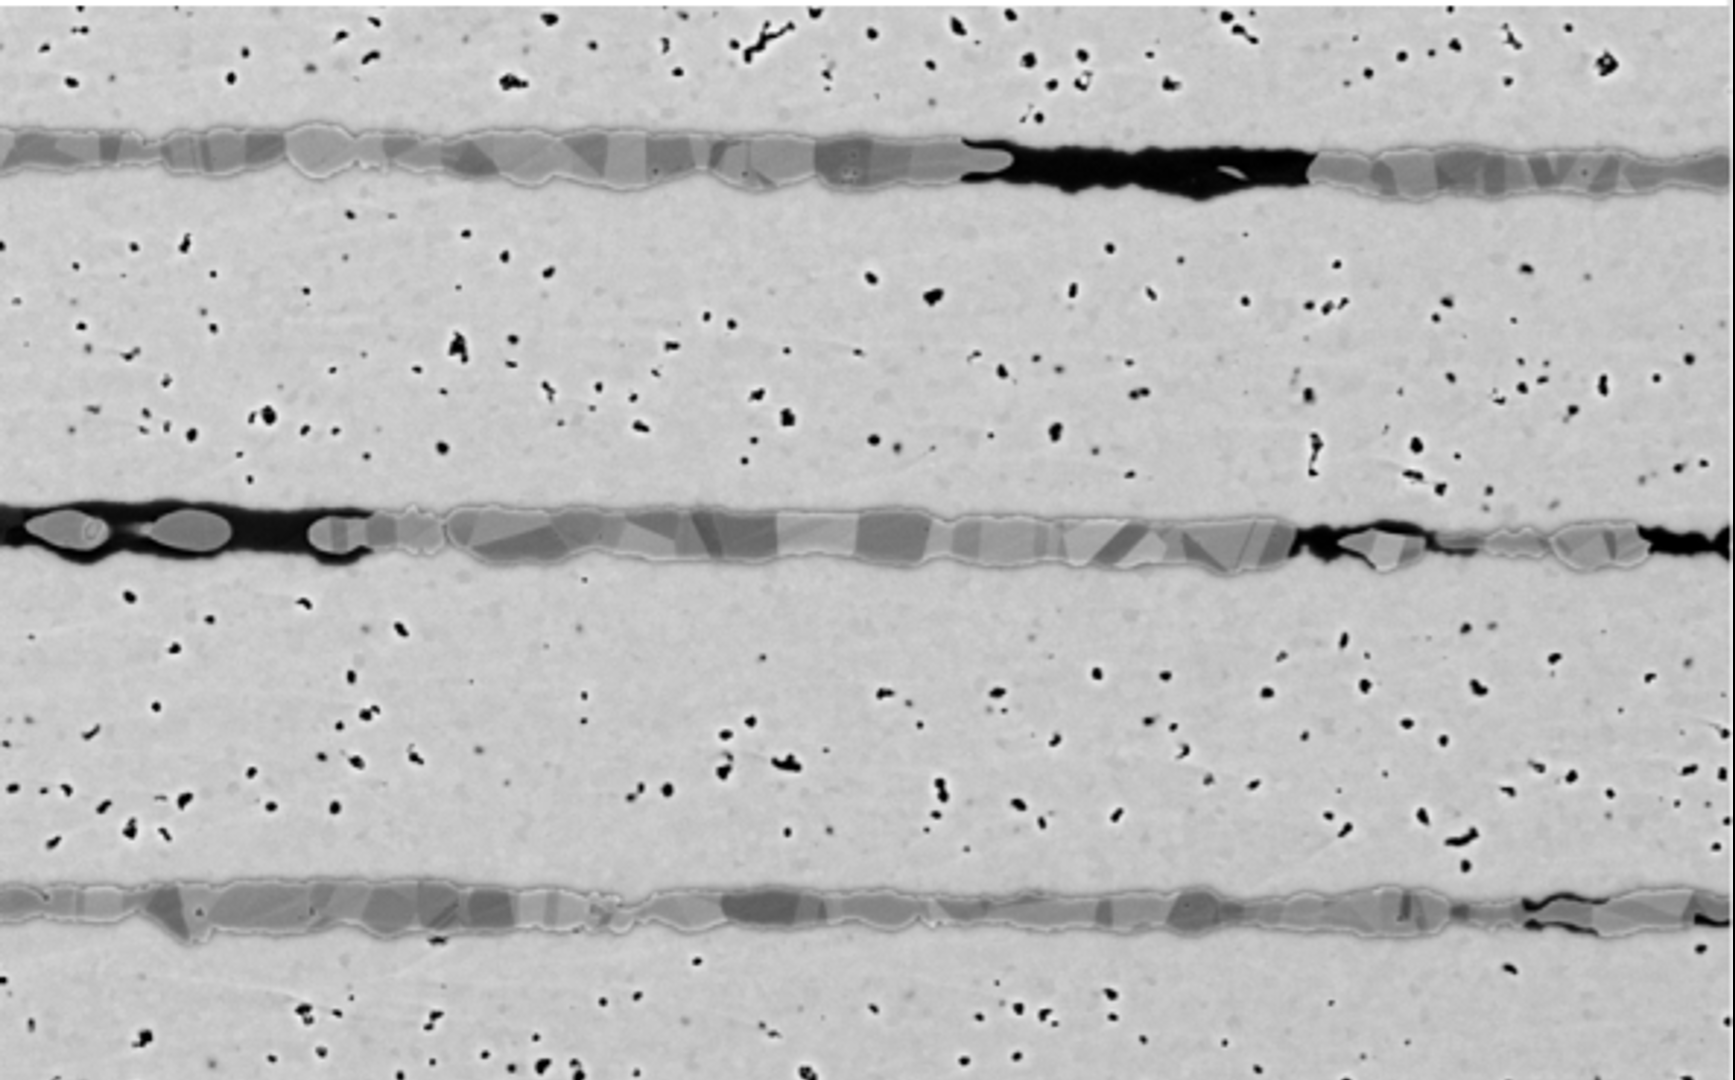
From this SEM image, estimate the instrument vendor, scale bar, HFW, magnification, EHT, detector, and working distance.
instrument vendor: Thermo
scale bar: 15000 nm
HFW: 51.9 µm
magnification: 10 K X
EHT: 10 kV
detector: BSD Full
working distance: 10.568 mm